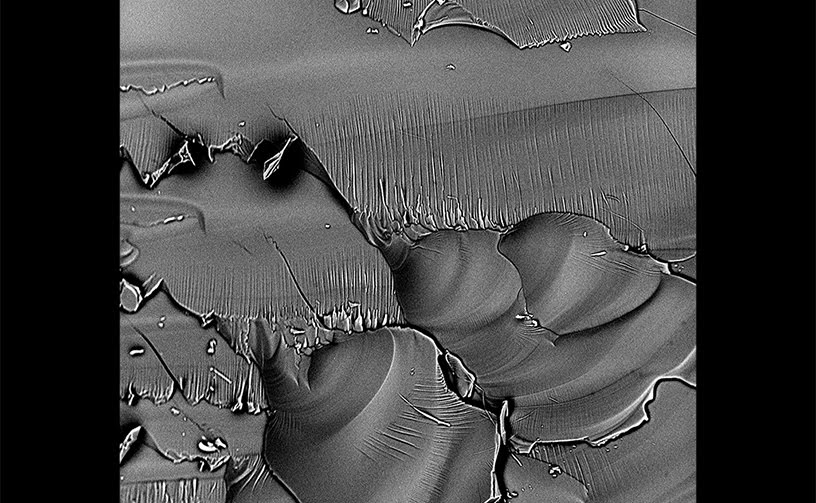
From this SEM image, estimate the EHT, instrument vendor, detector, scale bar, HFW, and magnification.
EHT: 10 kV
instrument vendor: other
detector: BSD Full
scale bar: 50000 nm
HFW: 255 µm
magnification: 1.05 K X